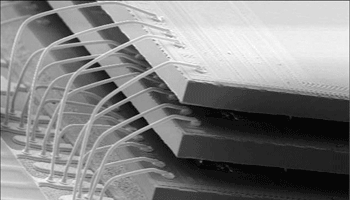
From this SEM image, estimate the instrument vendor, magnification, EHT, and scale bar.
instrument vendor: JEOL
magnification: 0.035 K X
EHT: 15 kV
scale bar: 500000 nm